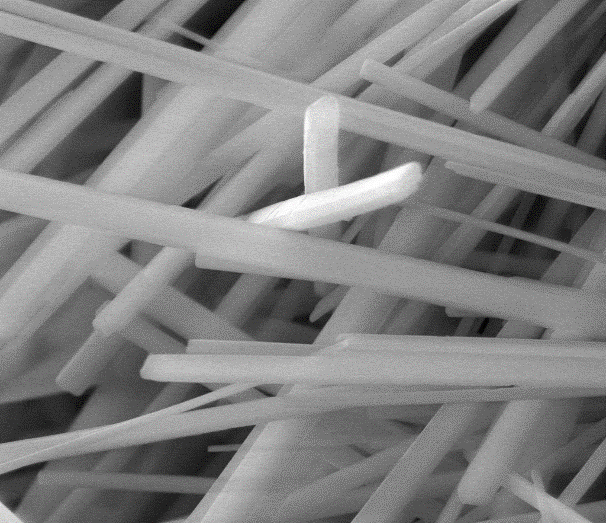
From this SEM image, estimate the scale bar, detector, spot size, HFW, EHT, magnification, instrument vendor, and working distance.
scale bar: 10000 nm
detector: LFD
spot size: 3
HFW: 29.8 µm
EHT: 20 kV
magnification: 10 K X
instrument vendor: FEI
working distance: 11.2 mm